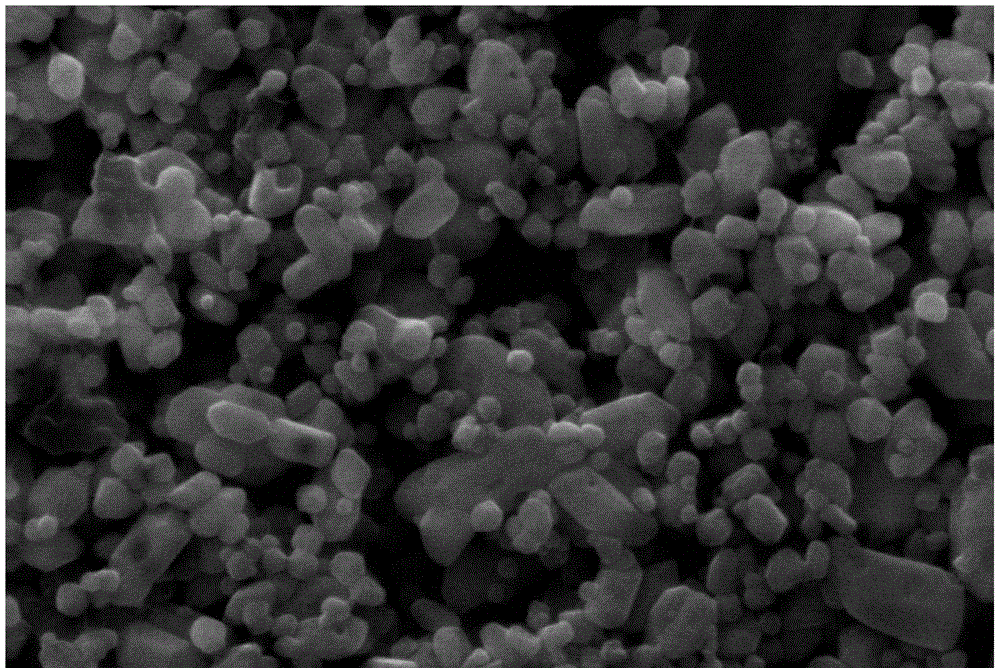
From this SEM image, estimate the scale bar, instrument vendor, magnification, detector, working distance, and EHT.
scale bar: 200 nm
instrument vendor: Zeiss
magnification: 20 K X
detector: SE1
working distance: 6 mm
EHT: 15 kV